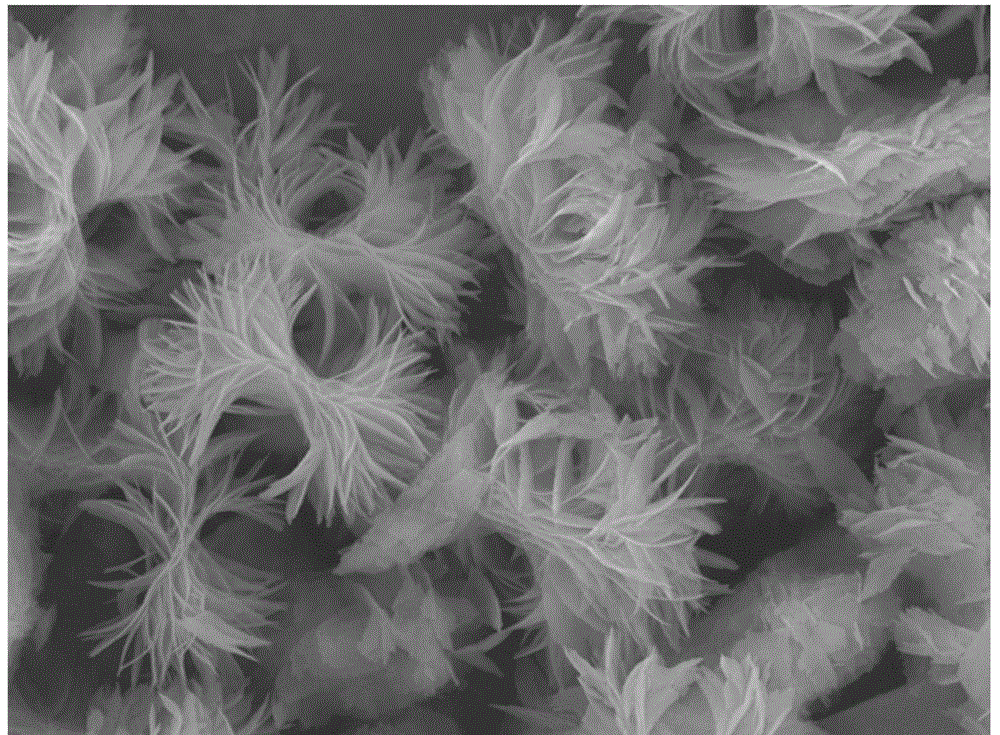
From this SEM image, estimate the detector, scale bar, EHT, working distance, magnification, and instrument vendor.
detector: LED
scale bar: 1000 nm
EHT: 15 kV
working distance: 9.8 mm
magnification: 4 K X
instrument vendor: JEOL